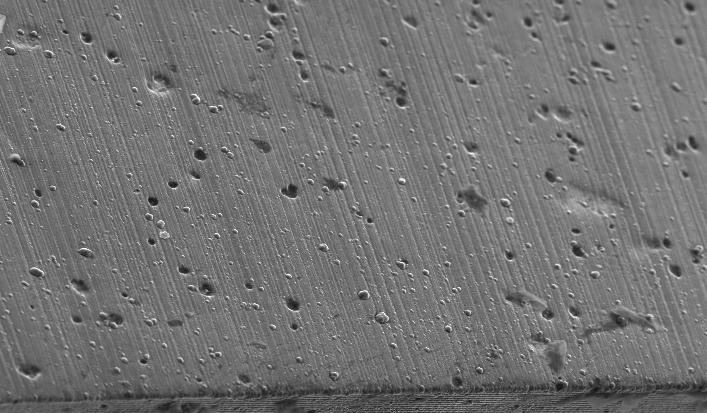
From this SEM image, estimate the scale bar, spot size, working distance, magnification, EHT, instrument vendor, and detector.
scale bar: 100000 nm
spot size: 5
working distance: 11.3 mm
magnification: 0.2 K X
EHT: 20 kV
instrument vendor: FEI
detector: SE